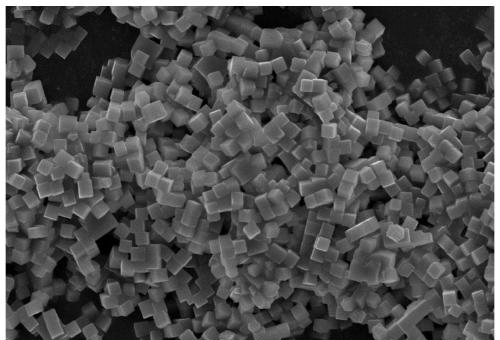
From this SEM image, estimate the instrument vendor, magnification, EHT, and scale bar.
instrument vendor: JEOL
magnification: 1.5 K X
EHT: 15 kV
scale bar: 10000 nm